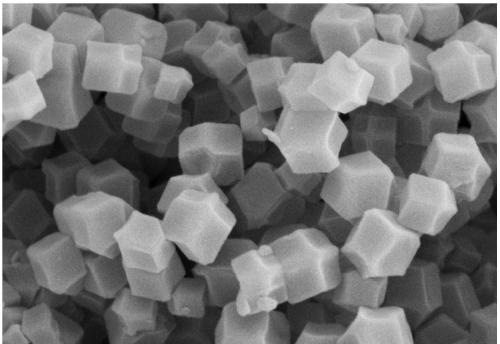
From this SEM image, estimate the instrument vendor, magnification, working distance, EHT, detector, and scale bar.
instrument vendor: Zeiss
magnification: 50 K X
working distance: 5.6 mm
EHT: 5 kV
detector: SE2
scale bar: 200 nm